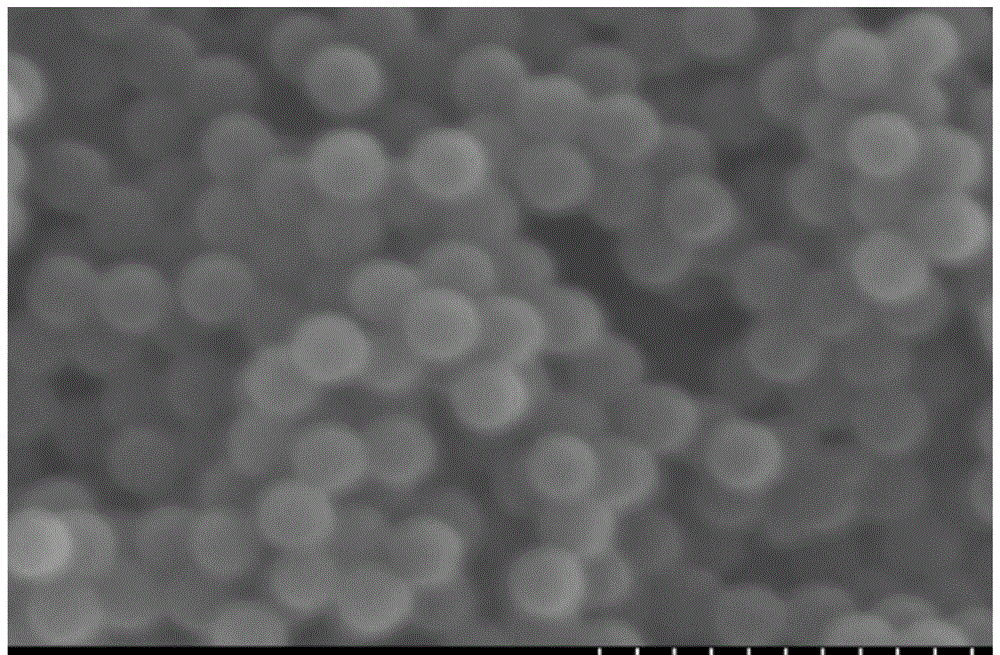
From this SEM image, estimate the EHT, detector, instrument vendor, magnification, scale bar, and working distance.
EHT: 15 kV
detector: SE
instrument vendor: Hitachi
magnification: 16 K X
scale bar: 3000 nm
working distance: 10.9 mm